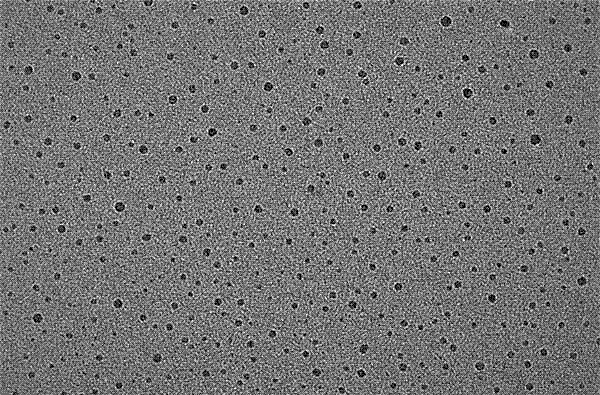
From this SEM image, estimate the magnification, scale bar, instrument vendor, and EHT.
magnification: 150 K X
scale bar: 50 nm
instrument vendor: JEOL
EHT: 200 kV